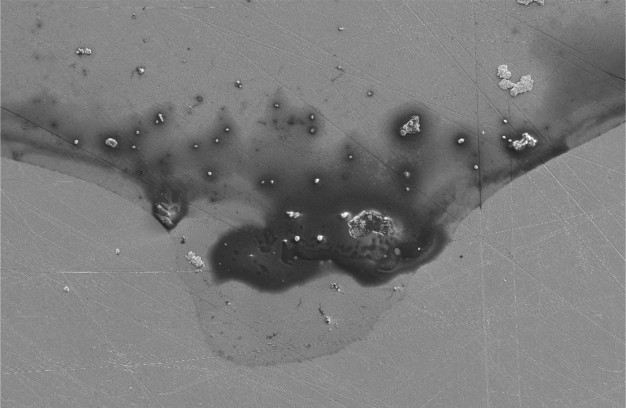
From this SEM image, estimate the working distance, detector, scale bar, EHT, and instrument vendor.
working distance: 11.4 mm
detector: SE2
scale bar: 20000 nm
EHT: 5 kV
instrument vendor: Zeiss